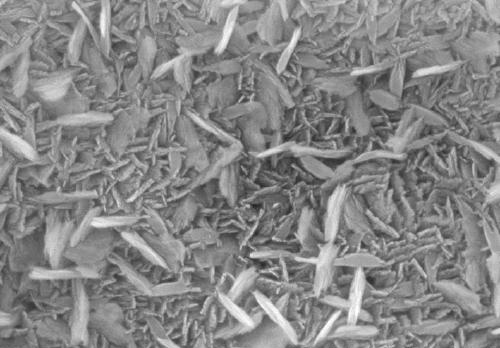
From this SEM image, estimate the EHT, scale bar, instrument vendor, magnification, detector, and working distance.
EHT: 15 kV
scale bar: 500 nm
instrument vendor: Hitachi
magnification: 100 K X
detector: SE(U)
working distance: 15.3 mm